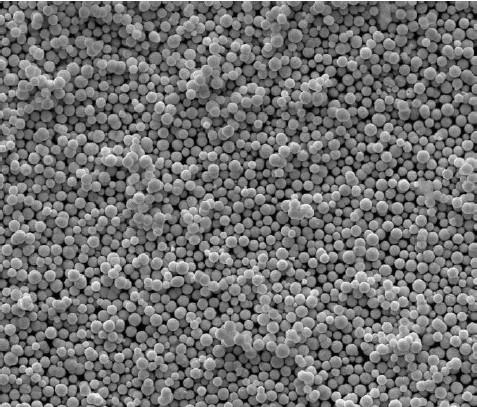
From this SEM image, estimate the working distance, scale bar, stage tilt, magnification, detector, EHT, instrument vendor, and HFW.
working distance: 8.7 mm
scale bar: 20000 nm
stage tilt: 0°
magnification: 5 K X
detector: ETD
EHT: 20 kV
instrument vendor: FEI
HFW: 59.7 µm